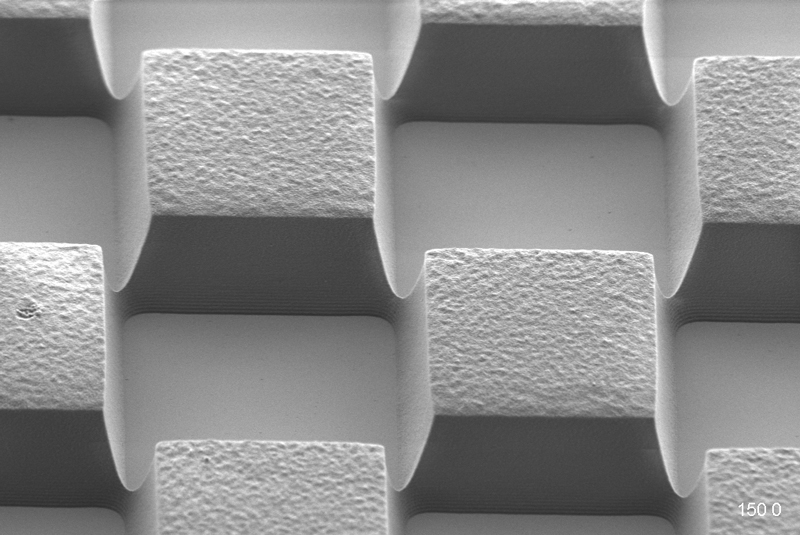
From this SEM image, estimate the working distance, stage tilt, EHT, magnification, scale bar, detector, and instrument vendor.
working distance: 7.3 mm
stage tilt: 45°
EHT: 1 kV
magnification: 4.03 K X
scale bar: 2000 nm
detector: HE-SE2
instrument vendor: Zeiss